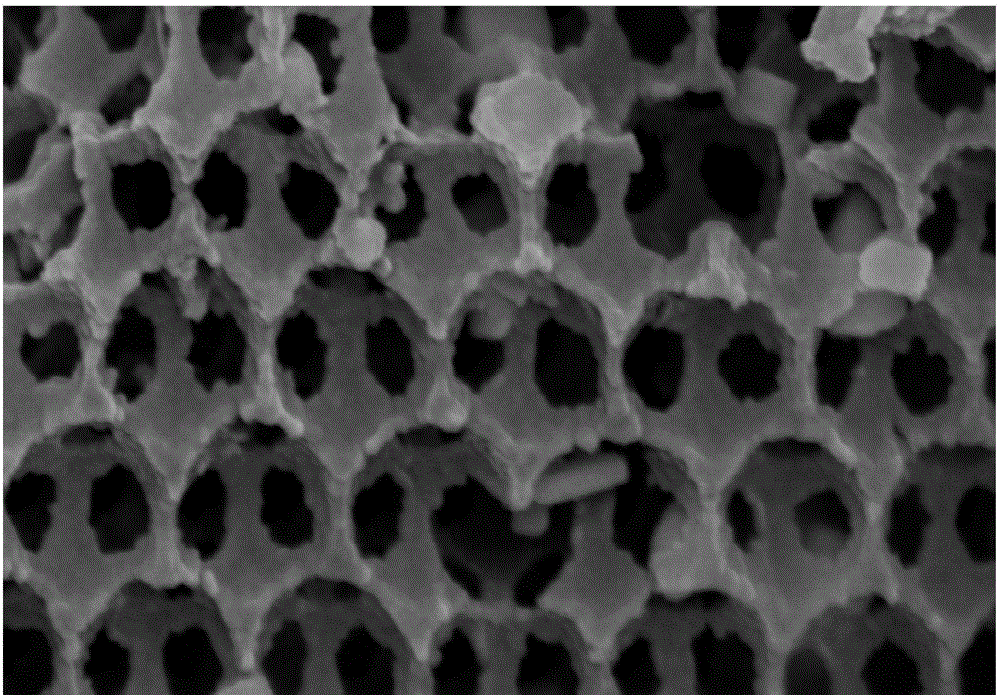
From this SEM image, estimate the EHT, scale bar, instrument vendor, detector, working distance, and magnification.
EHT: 15 kV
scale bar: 500 nm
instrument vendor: Hitachi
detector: SE(M)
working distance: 7.1 mm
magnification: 70 K X